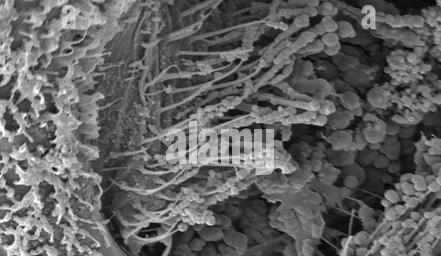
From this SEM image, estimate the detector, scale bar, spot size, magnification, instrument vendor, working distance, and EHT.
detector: SE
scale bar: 20000 nm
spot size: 3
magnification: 1.601 K X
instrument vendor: FEI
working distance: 6.1 mm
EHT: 10 kV